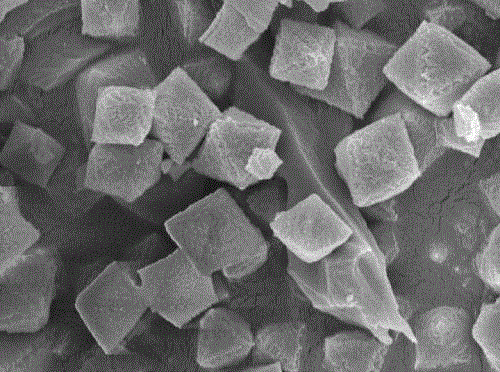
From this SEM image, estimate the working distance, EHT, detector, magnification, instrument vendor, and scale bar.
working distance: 6.8 mm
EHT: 5 kV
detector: SEI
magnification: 15 K X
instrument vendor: JEOL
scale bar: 1000 nm